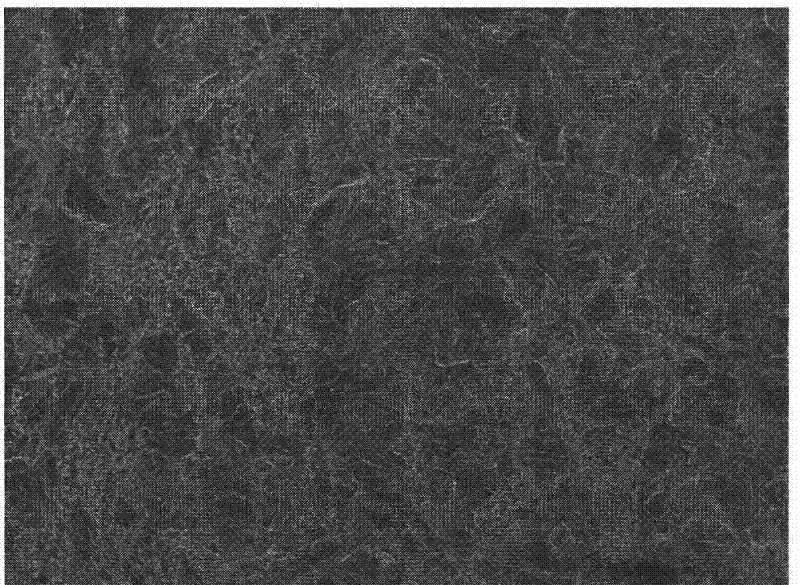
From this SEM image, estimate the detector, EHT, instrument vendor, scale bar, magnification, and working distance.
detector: SEI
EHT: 5 kV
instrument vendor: JEOL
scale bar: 10000 nm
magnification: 0.5 K X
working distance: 8.5 mm